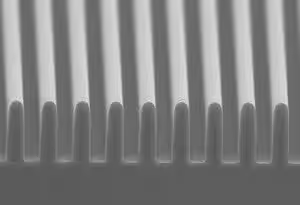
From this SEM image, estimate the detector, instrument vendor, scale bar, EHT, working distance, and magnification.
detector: SE(L)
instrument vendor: Hitachi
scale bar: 5000 nm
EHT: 5 kV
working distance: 7.6 mm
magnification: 10 K X